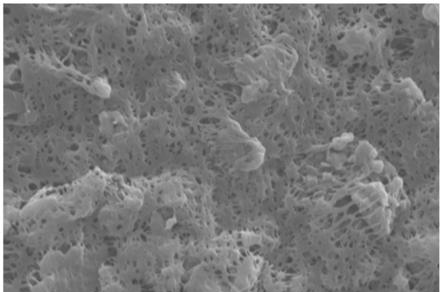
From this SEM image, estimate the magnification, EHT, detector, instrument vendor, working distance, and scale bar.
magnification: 10 K X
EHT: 20 kV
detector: SE1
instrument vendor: Zeiss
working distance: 7 mm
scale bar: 1000 nm